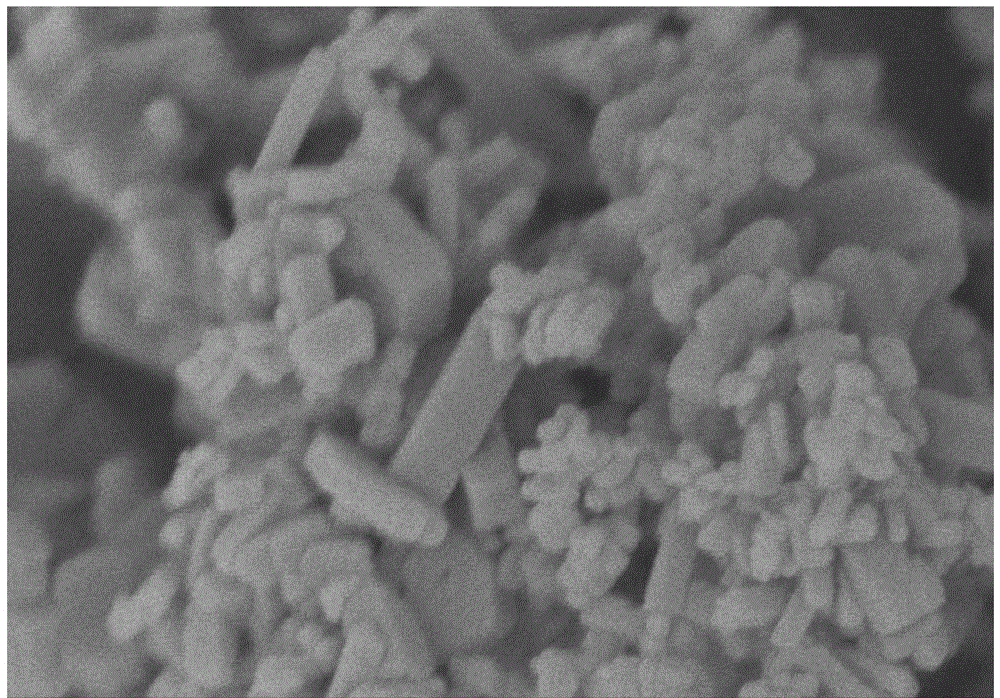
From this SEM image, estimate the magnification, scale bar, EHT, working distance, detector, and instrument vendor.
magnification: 100 K X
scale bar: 500 nm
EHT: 3 kV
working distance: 5 mm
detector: SE(U)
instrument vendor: Hitachi